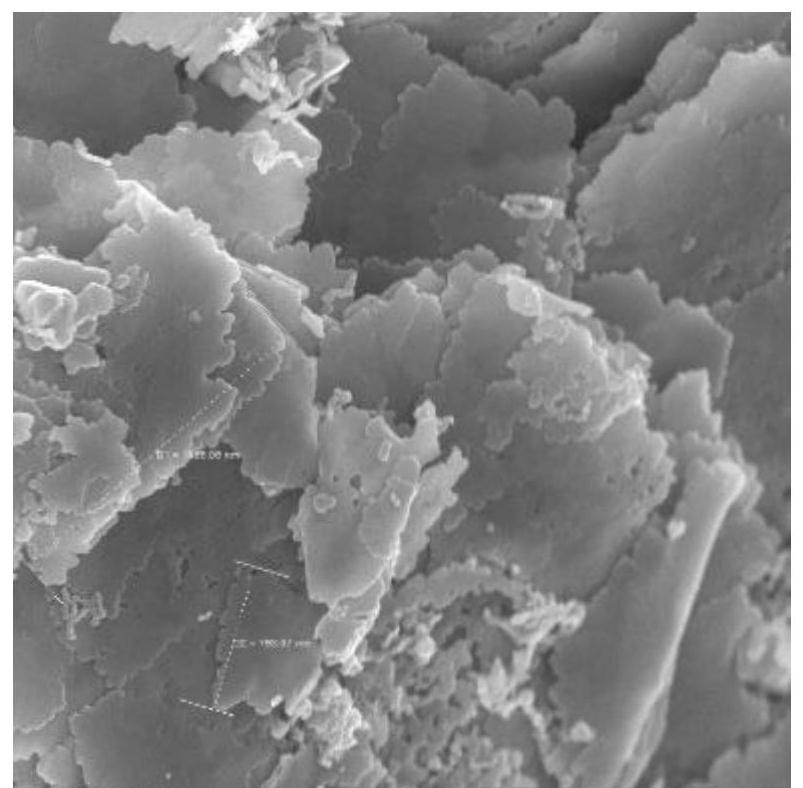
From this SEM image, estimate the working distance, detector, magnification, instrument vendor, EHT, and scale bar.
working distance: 4.92 mm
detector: In-Beam SE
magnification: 50 K X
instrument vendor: Tescan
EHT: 10 kV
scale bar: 1000 nm